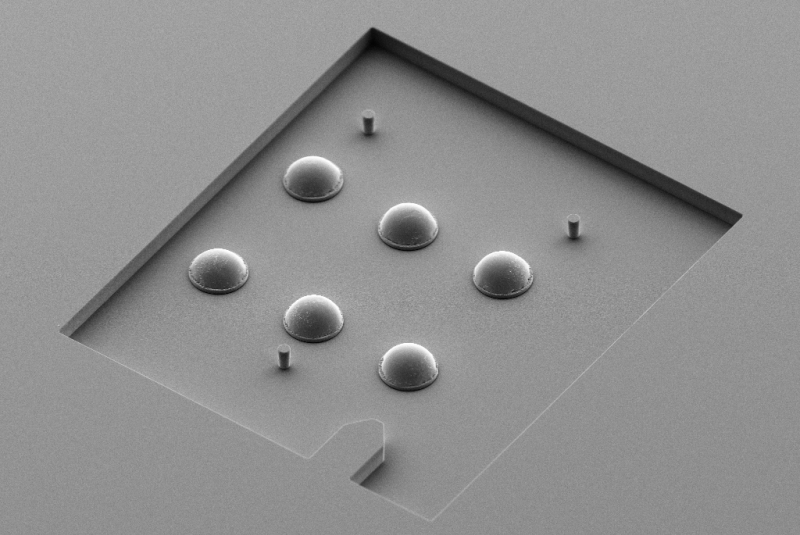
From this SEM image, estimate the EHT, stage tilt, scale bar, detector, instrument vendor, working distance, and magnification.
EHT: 5 kV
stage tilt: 45°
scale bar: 20000 nm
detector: SE2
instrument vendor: Zeiss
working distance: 8.2 mm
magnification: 0.35 K X